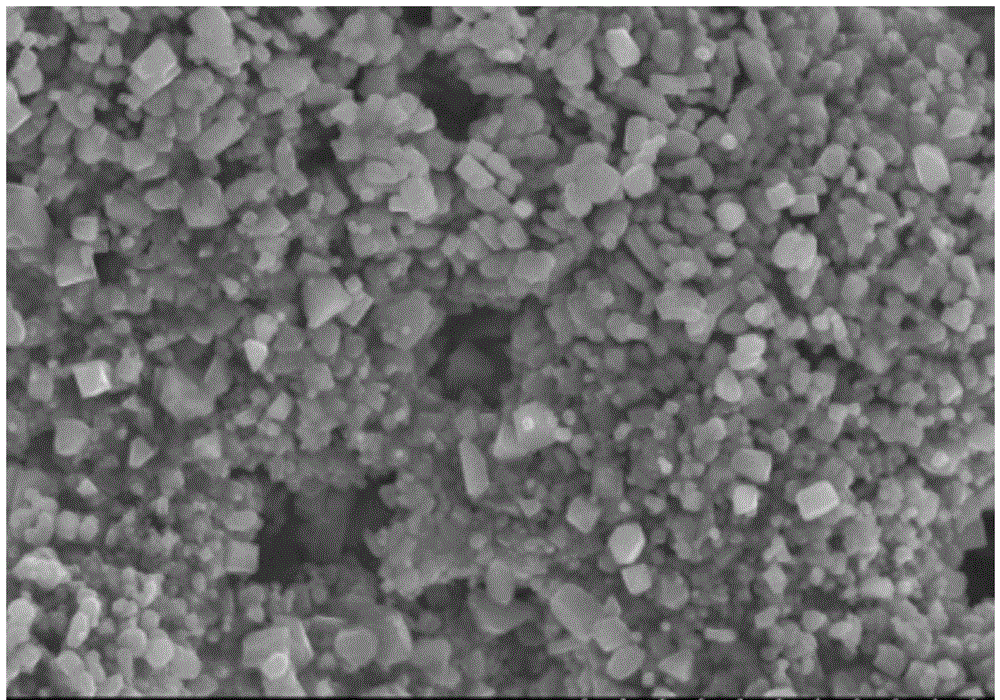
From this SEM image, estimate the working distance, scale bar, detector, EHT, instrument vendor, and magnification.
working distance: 10.1 mm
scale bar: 10000 nm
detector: SE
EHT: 15 kV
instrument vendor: Hitachi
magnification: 5 K X